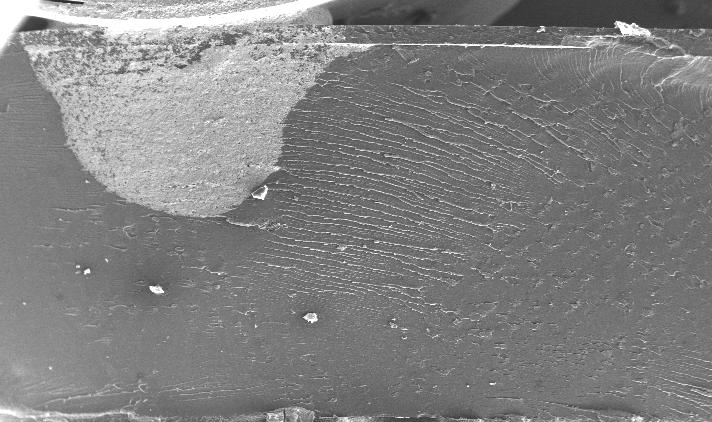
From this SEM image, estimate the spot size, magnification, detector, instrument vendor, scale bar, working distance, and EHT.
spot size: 6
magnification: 0.05 K X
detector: SE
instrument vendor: FEI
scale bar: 1000000 nm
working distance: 10.4 mm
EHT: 20 kV